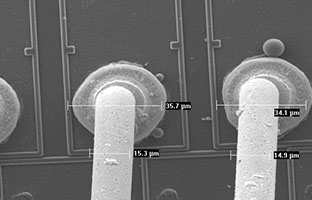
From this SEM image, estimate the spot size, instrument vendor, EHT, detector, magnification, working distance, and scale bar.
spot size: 3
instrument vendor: FEI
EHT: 10 kV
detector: SE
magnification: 0.699 K X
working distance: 9.3 mm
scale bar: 20000 nm